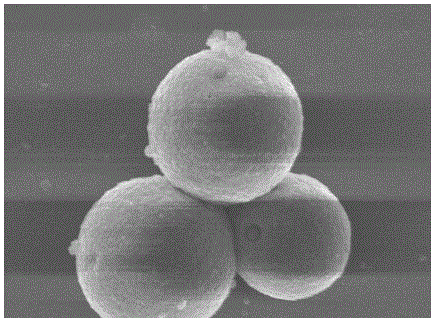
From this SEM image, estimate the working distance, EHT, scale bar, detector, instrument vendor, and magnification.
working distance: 7.4 mm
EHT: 8 kV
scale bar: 1000 nm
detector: SEI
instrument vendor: JEOL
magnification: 15 K X